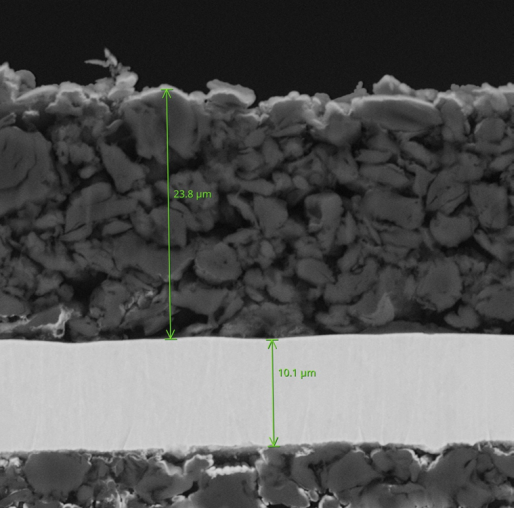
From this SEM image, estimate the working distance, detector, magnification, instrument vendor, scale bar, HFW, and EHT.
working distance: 4.972 mm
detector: Image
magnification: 5.5 K X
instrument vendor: Thermo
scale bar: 10000 nm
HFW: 48.8 µm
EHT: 15 kV